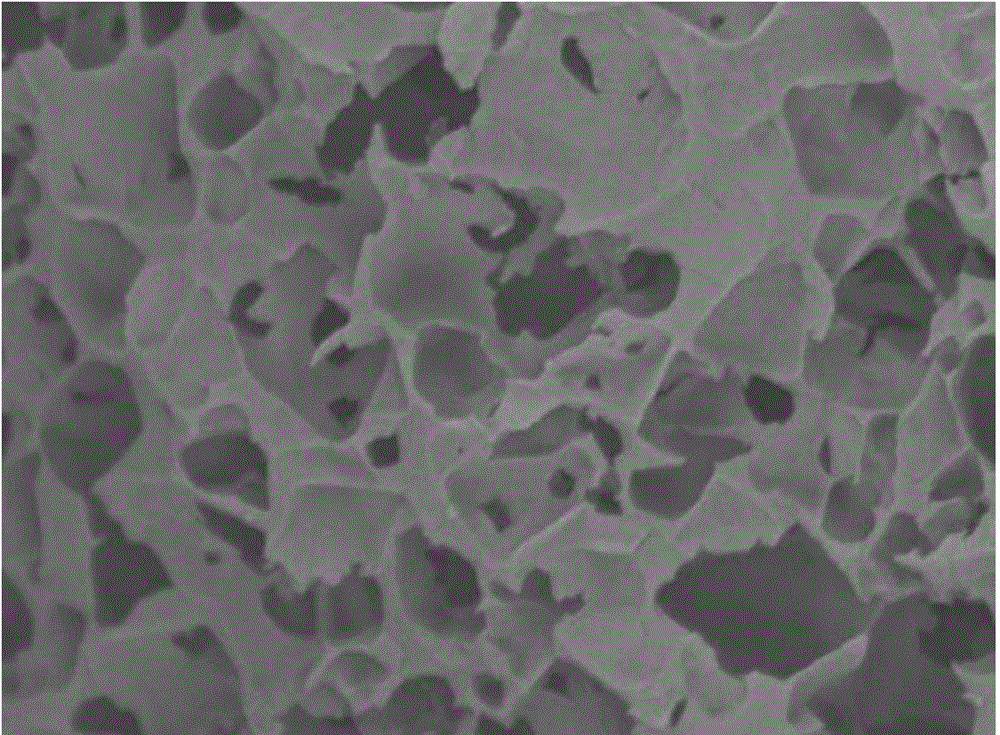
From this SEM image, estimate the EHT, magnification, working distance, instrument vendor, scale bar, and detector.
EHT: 3 kV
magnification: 10 K X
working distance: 8 mm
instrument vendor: JEOL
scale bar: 1000 nm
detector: LEI